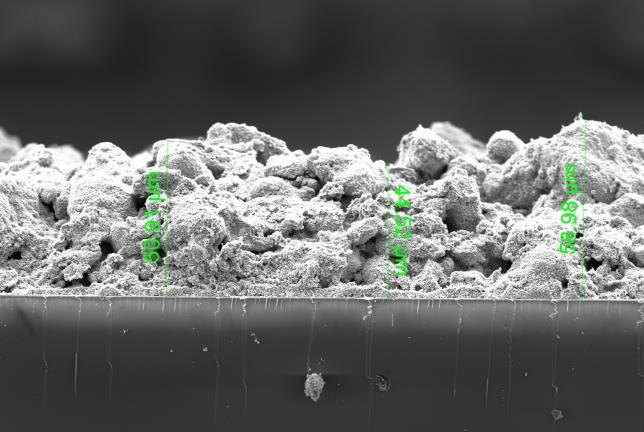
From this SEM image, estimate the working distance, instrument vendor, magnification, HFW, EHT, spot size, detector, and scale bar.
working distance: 9.5 mm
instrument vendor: FEI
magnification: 1.903 K X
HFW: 218 µm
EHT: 5 kV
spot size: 3.5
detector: ETD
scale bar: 50000 nm